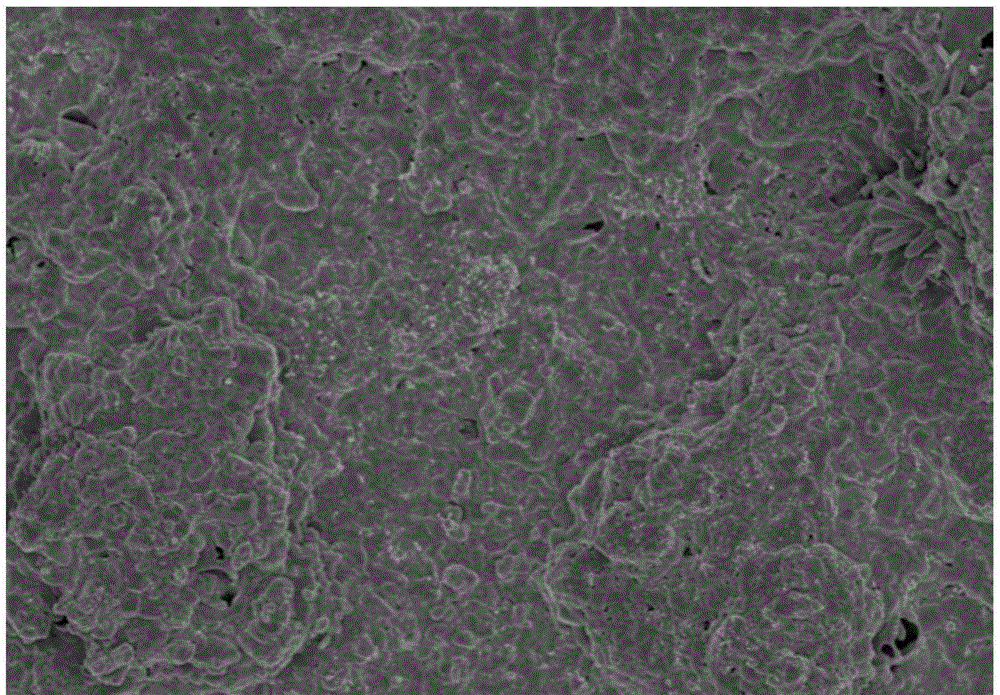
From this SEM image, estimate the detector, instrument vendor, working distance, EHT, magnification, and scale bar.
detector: SE(U)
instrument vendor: Hitachi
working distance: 7.6 mm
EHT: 5 kV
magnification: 5 K X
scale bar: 10000 nm